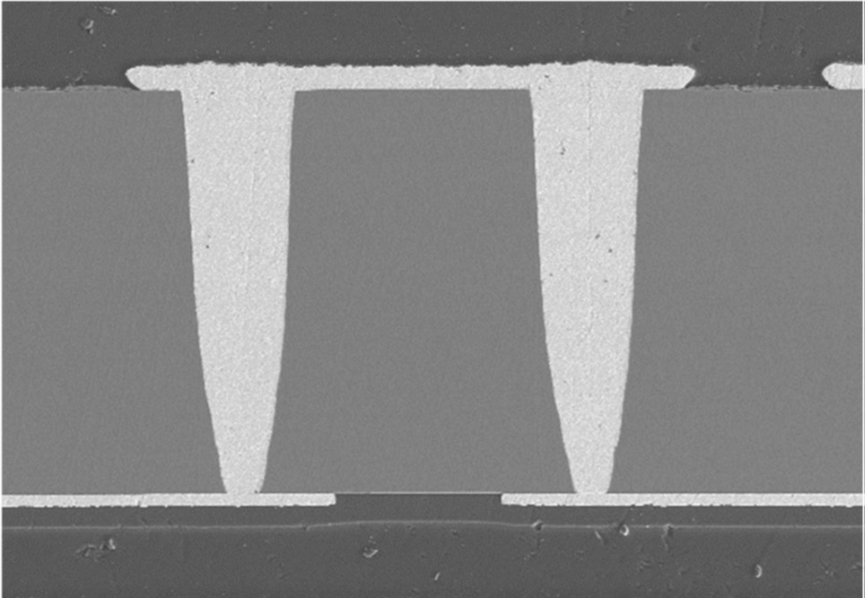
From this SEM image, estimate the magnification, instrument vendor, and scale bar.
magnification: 0.5 K X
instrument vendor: other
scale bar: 100000 nm